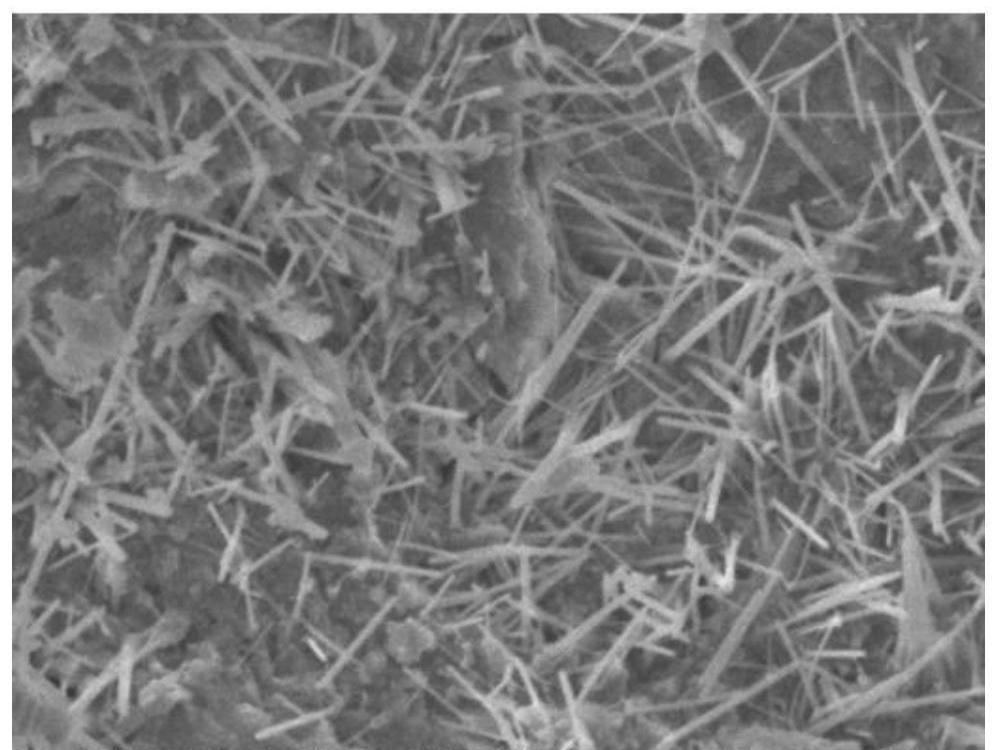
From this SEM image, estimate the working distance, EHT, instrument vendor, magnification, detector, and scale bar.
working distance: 10.6 mm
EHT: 5 kV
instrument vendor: JEOL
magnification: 10 K X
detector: SED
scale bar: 1000 nm